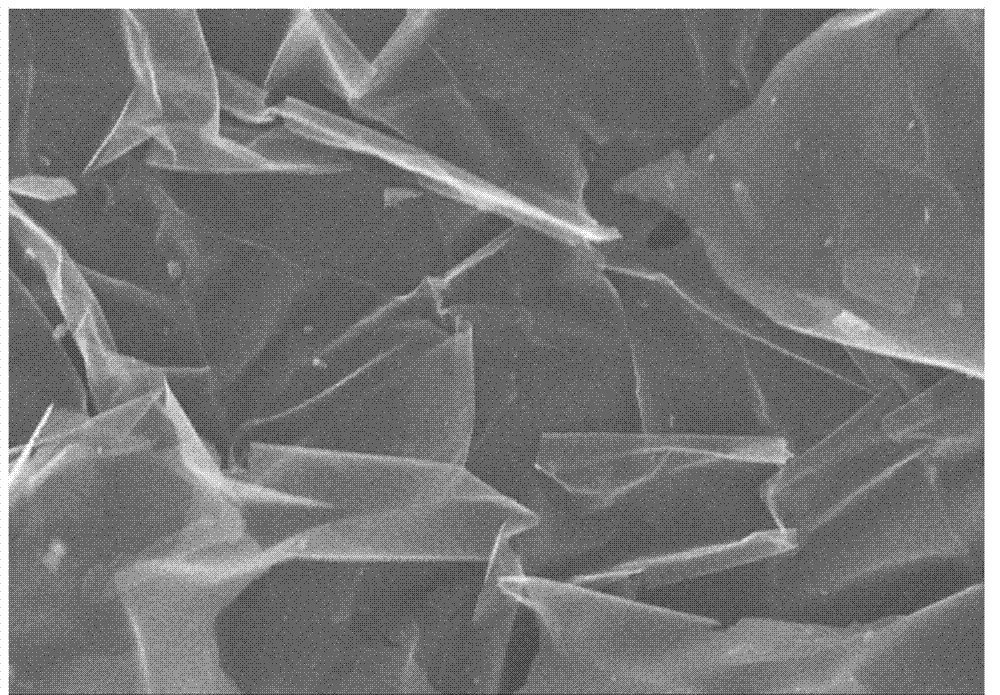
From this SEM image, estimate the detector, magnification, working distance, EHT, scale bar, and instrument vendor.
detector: SE(M)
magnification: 5 K X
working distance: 9.8 mm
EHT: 10 kV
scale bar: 10000 nm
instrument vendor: Hitachi